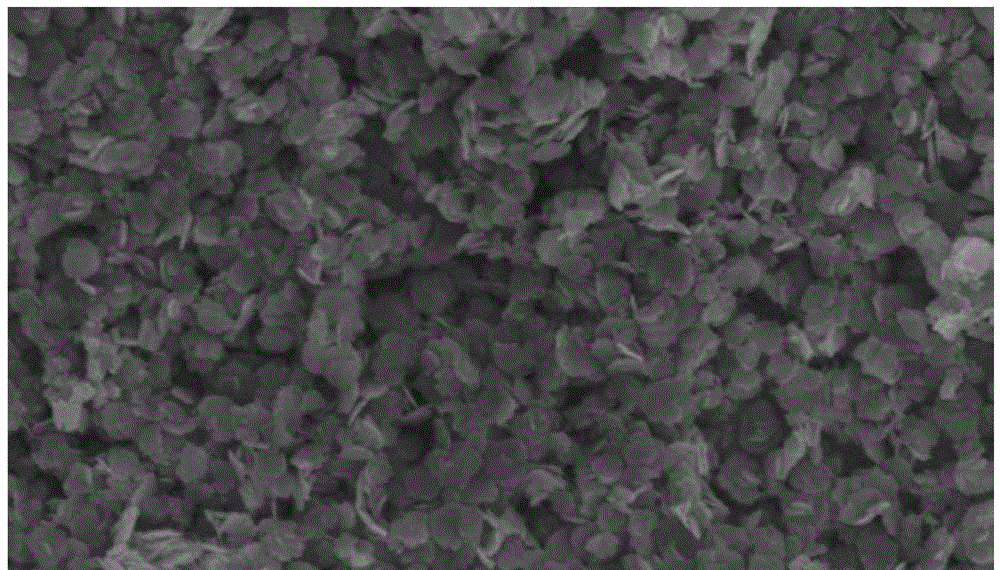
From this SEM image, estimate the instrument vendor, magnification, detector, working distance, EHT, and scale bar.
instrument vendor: Hitachi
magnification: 20 K X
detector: SE(M)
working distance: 9.9 mm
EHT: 5 kV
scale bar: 2000 nm